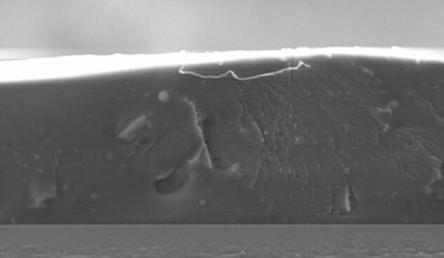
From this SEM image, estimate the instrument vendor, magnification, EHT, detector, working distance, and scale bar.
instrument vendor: Zeiss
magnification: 0.4 K X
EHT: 20 kV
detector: SE2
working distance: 10 mm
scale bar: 10000 nm